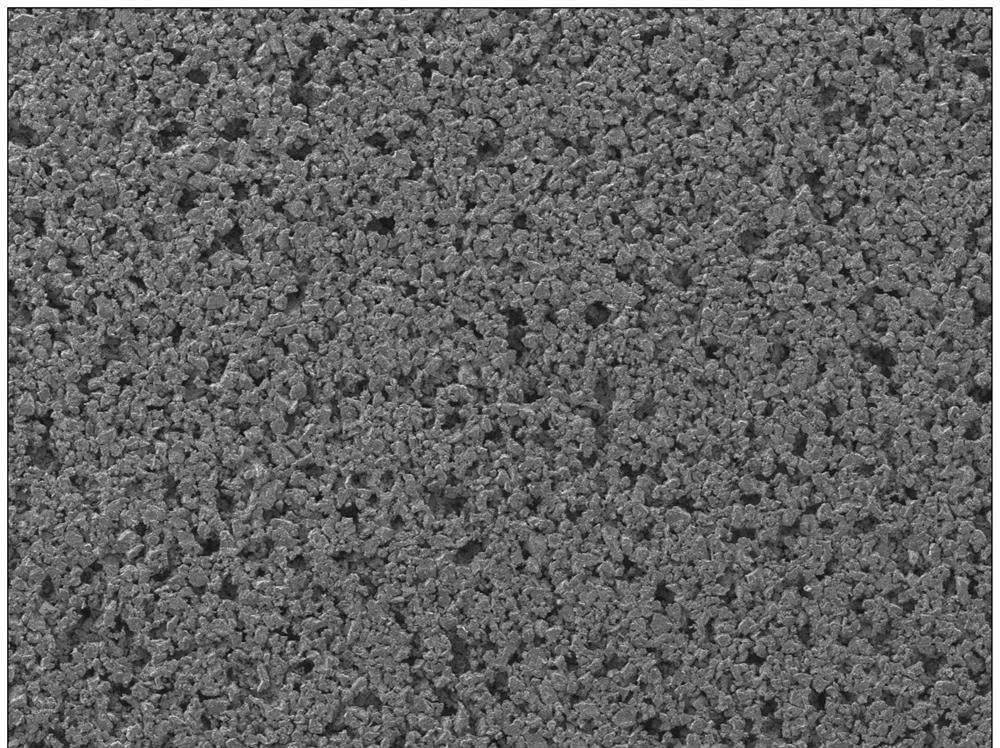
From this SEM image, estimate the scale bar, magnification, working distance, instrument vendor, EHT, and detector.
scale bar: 100000 nm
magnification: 0.05 K X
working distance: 8.1 mm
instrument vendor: JEOL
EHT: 5 kV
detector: LEI